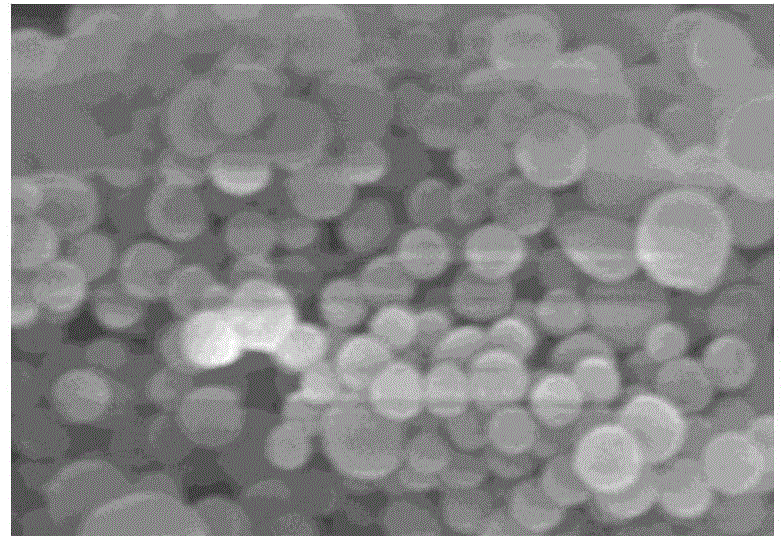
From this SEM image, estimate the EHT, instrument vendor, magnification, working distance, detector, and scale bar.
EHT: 5 kV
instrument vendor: Hitachi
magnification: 50 K X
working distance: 9.4 mm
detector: SE(M)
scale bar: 1000 nm